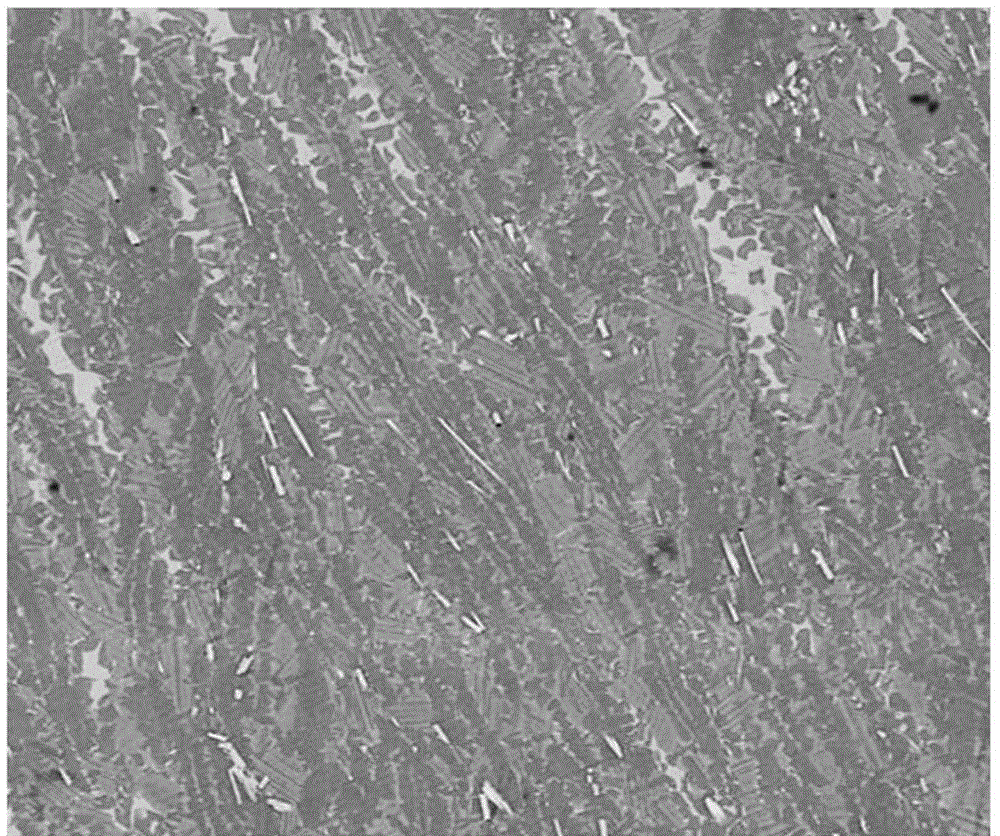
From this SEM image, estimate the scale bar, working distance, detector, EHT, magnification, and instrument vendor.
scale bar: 100000 nm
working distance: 10.6 mm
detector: Z Cont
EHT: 20 kV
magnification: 1 K X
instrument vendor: FEI